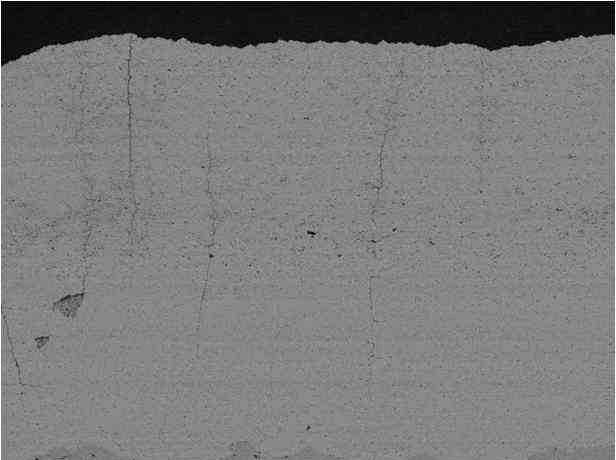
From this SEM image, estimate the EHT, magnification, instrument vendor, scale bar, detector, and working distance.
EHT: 15 kV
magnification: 0.15 K X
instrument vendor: JEOL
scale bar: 100000 nm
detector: COMPO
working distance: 8 mm